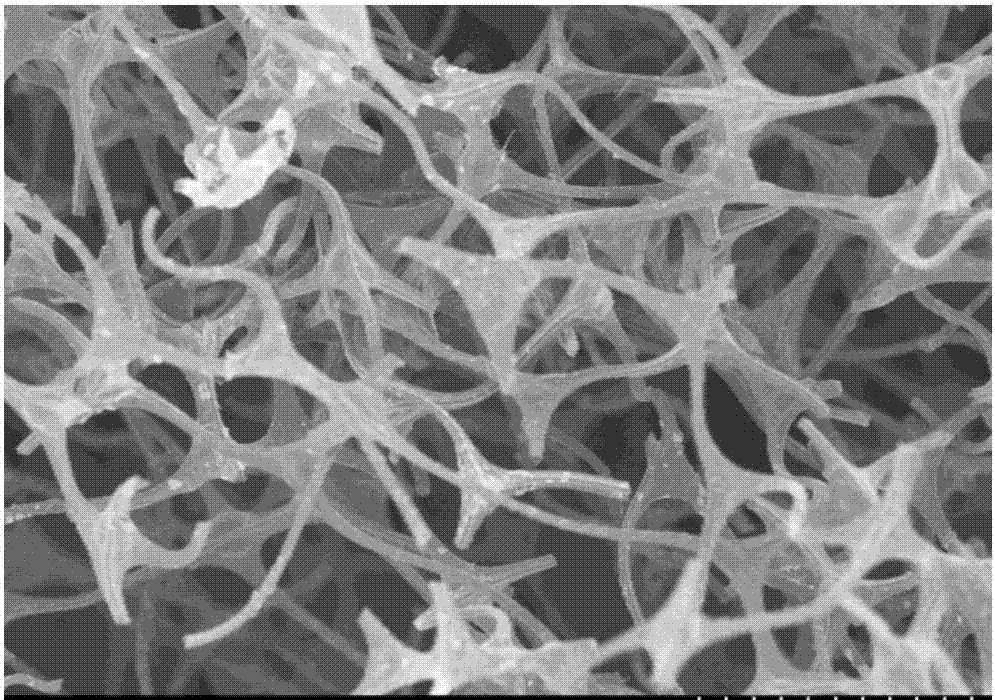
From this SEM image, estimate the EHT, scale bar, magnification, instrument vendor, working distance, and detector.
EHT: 3 kV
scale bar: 50000 nm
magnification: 0.7 K X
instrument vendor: Hitachi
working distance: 8.6 mm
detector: SE(M)